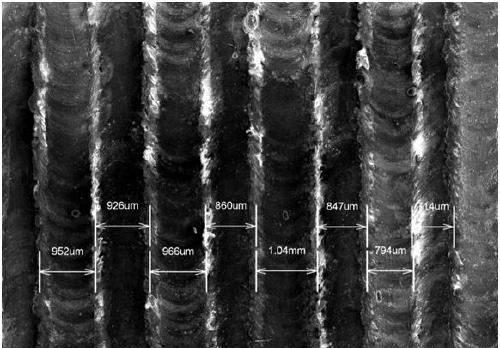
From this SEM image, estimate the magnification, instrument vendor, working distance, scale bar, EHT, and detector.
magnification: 0.015 K X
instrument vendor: Hitachi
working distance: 41.9 mm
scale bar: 3000000 nm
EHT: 15 kV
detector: SE